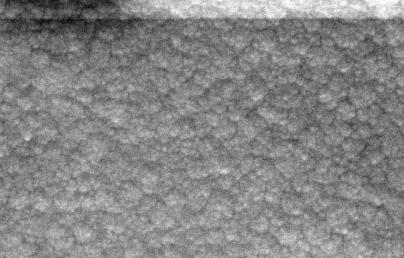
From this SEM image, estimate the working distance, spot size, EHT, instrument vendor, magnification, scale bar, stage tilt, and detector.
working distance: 16 mm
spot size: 350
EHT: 20 kV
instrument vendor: Zeiss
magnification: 150 K X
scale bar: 1000 nm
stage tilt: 0°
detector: SE1